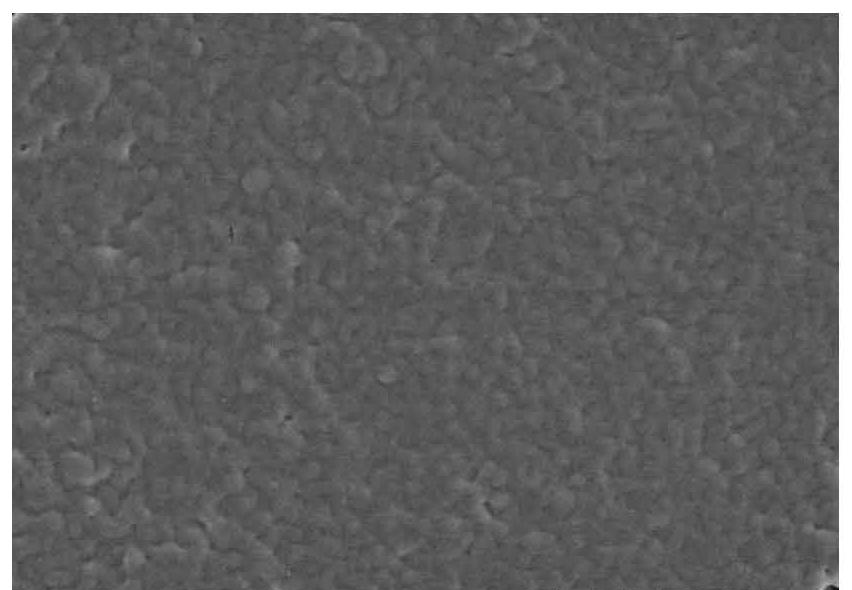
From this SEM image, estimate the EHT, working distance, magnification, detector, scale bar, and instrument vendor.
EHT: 3 kV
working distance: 10.1 mm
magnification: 10 K X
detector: SE(M)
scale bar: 5000 nm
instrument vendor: Hitachi